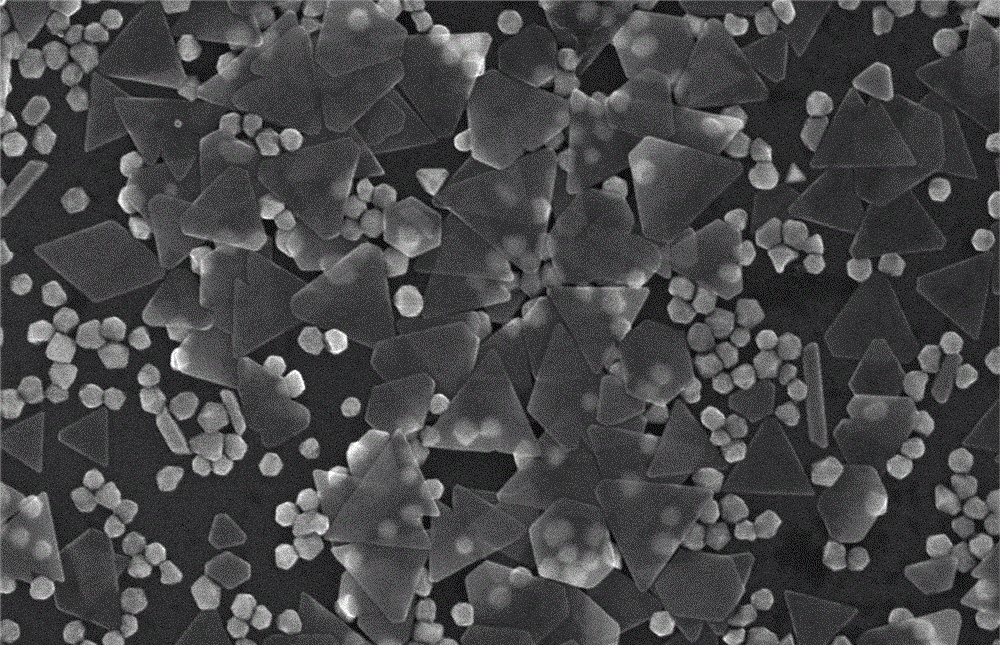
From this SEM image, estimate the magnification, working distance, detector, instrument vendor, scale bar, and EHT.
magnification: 35 K X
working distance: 8.9 mm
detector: SE(U)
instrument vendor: Hitachi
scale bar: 1000 nm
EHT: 10 kV